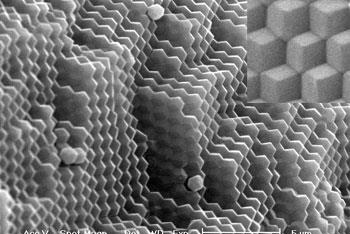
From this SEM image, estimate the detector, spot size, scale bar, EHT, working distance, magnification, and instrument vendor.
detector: SE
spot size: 4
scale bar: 5000 nm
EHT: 20 kV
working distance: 5.3 mm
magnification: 5 K X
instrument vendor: FEI